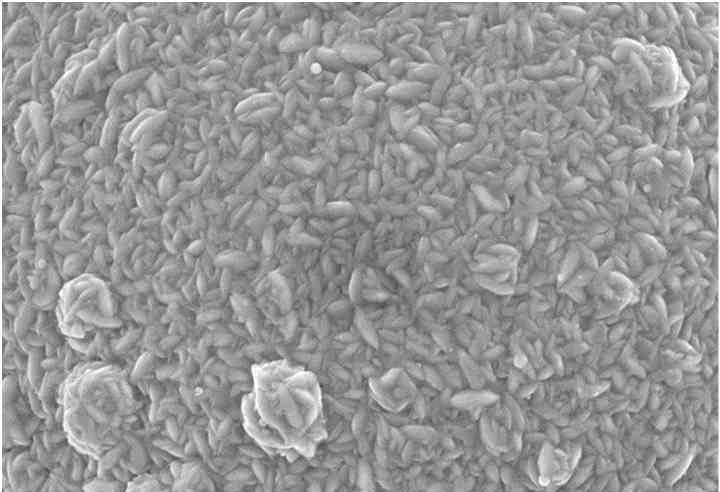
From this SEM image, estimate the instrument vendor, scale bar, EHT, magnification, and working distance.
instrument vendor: Zeiss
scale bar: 5000 nm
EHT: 15 kV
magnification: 5 K X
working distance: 18 mm